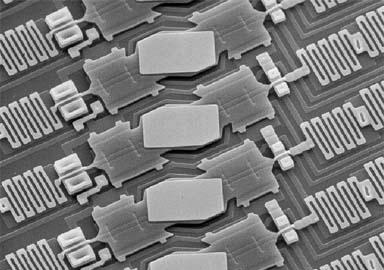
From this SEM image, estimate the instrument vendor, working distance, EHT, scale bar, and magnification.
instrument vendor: JEOL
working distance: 14 mm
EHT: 35 kV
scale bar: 10000 nm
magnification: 0.45 K X